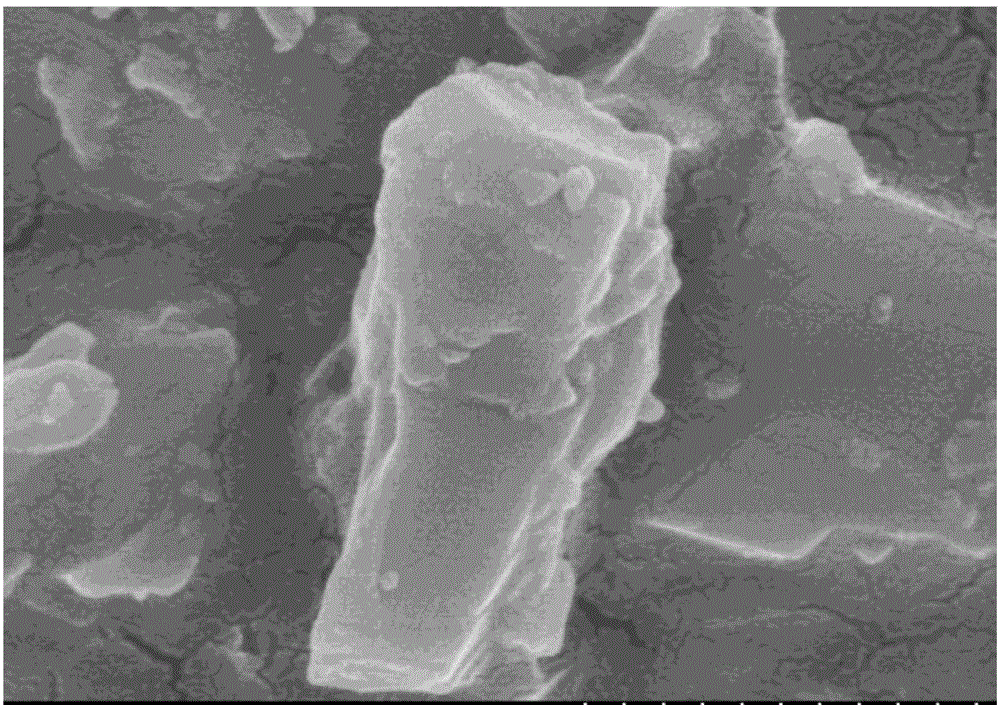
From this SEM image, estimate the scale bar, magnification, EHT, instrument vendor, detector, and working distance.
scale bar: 500 nm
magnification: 100 K X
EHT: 5 kV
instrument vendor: Hitachi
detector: SE(U)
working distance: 5.9 mm